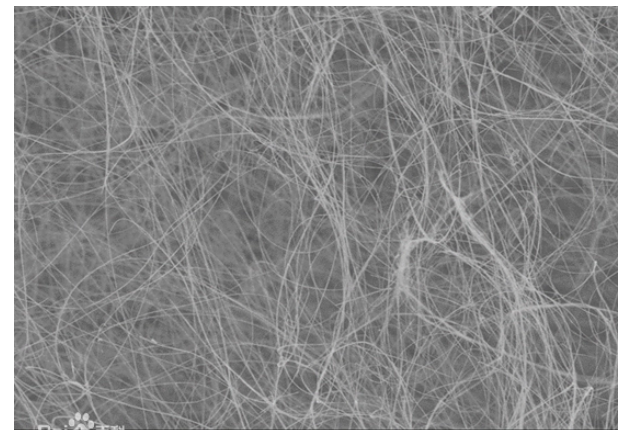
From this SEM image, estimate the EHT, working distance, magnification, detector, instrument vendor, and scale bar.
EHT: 5 kV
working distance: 8.3 mm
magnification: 1 K X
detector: SE(M)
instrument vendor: Hitachi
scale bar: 50000 nm